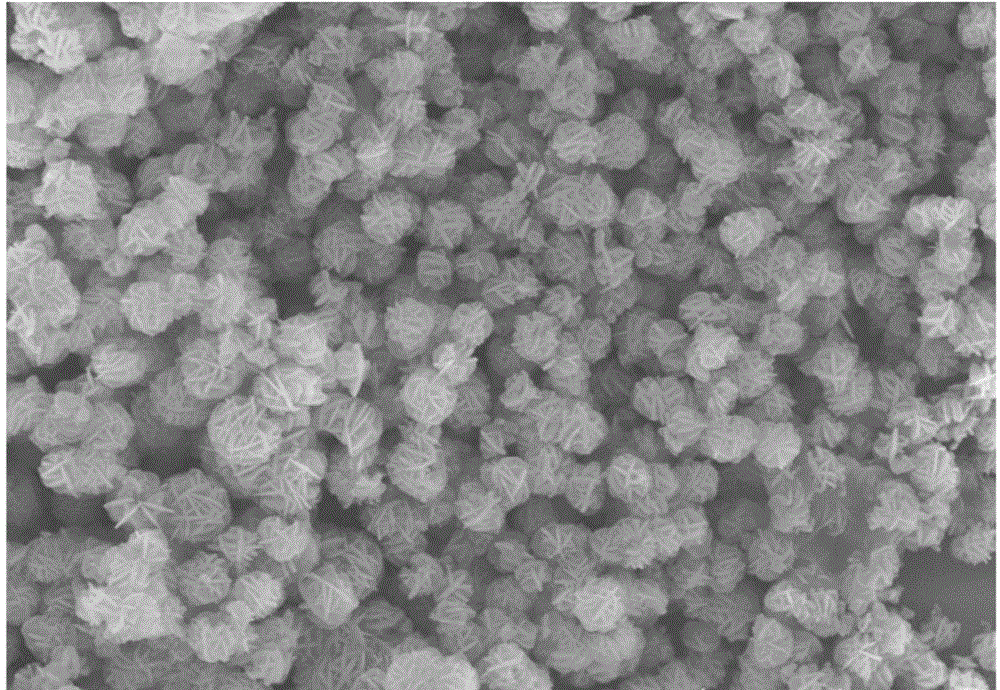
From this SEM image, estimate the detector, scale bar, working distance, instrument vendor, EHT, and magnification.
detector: SE(U)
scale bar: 10000 nm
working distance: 12.3 mm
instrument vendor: Hitachi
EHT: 15 kV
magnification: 3.5 K X